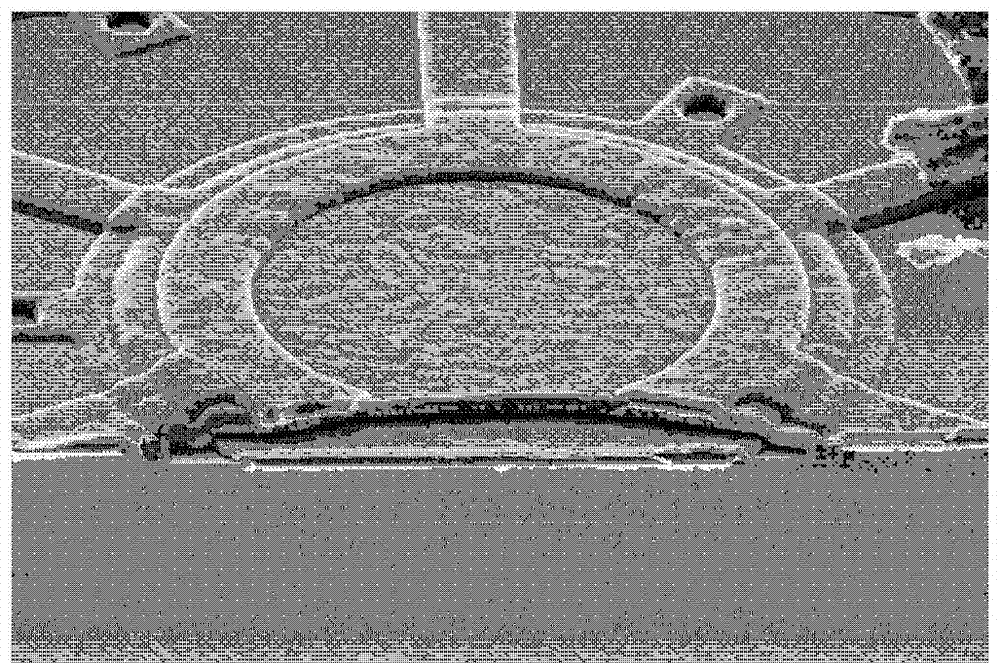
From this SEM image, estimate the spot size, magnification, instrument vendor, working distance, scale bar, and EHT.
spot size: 2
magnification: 6.5 K X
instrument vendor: FEI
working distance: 10.1 mm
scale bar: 10000 nm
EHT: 7 kV